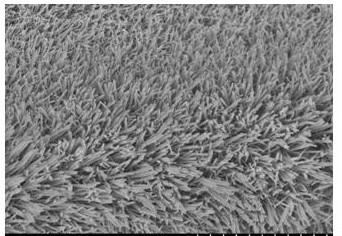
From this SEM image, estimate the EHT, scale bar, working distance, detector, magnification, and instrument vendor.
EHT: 5 kV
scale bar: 20000 nm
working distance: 10.7 mm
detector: SE(M)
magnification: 2.5 K X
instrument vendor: Hitachi